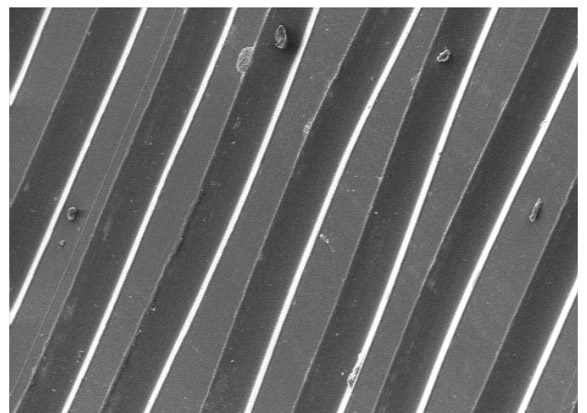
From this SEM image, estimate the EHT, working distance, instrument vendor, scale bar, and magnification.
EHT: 10 kV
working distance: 10 mm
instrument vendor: other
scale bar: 200000 nm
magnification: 0.2 K X